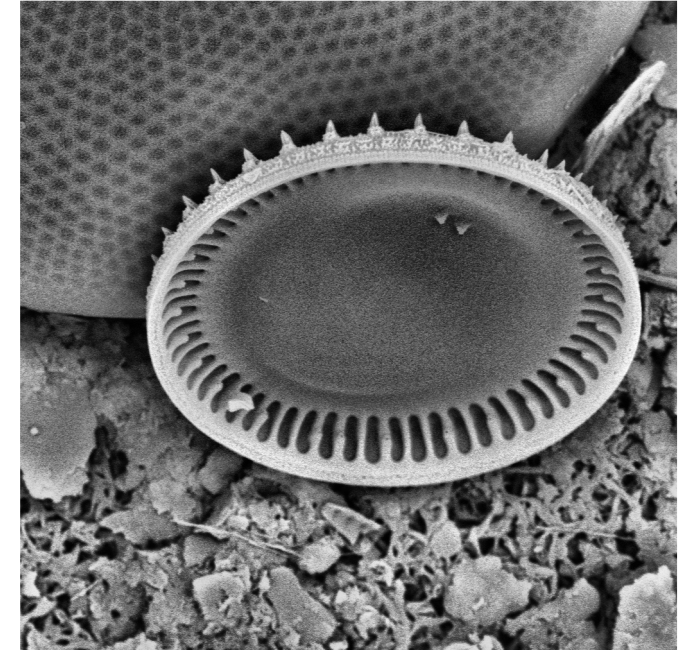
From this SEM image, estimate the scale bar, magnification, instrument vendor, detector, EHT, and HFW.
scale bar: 10000 nm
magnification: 8.3 K X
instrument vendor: Thermo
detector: BSD Full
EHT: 10 kV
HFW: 32.3 µm